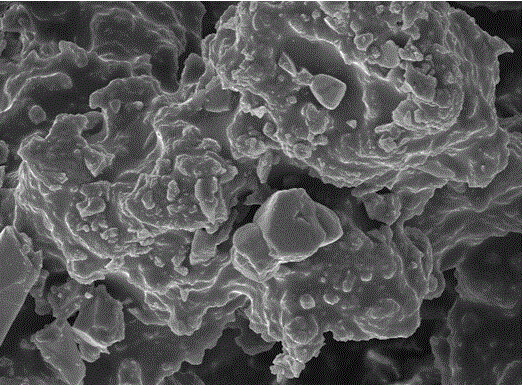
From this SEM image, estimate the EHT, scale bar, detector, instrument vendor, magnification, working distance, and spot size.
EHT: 10 kV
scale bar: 10000 nm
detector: SE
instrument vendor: FEI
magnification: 2 K X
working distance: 5.1 mm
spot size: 3